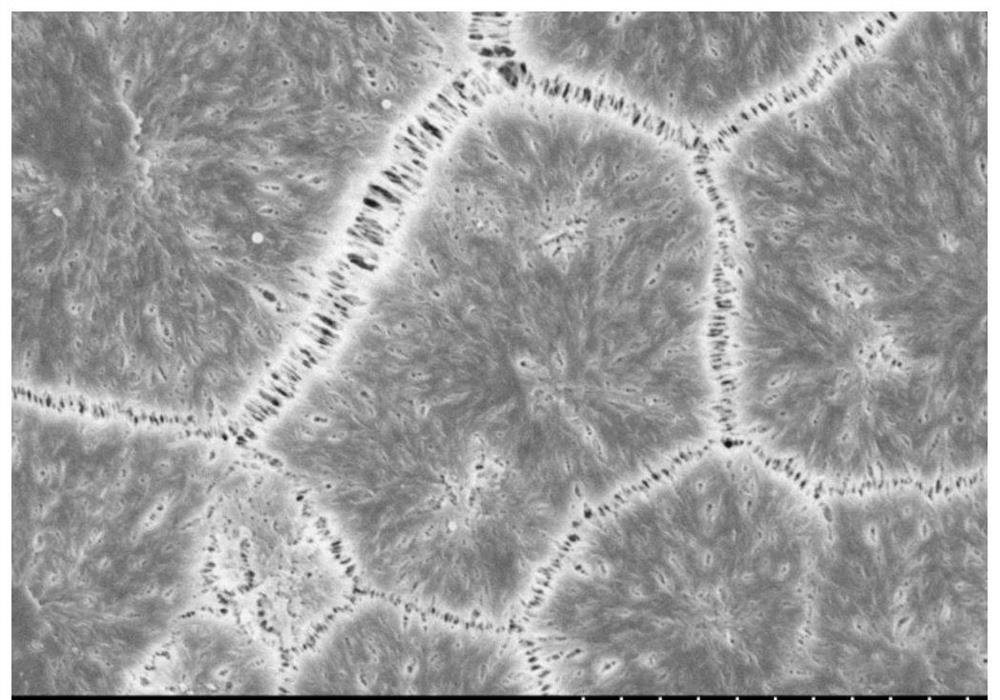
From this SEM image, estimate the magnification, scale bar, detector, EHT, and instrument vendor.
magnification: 5 K X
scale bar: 10000 nm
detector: SE(U)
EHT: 4 kV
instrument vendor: Hitachi